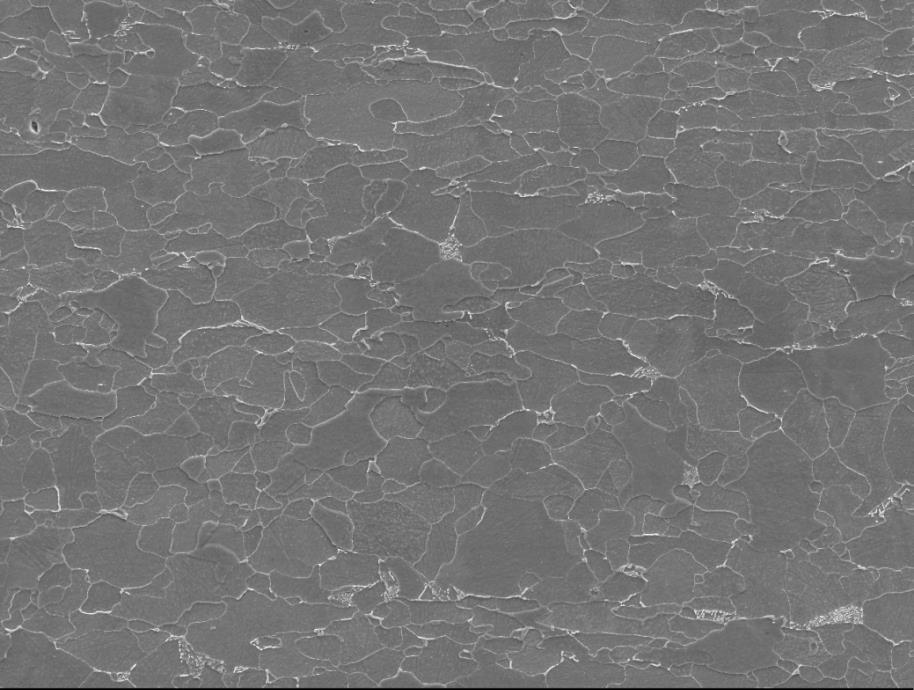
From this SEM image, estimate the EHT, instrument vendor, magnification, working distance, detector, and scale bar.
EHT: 15 kV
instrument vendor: JEOL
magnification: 1 K X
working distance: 9.9 mm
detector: SEI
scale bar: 10000 nm